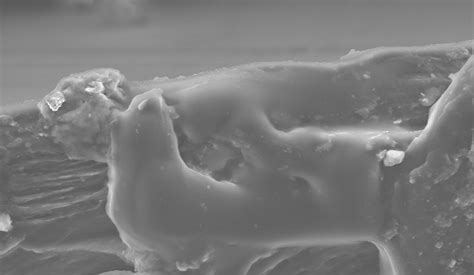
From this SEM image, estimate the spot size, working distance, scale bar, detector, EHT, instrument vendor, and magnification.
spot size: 2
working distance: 10.8 mm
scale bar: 10000 nm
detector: SE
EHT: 10 kV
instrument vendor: FEI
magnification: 2 K X